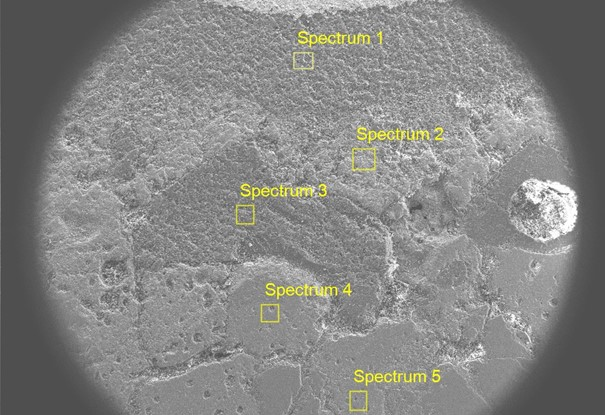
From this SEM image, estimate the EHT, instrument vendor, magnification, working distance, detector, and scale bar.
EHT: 15 kV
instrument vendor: other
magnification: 0.03 K X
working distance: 5.5 mm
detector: SE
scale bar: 800000 nm